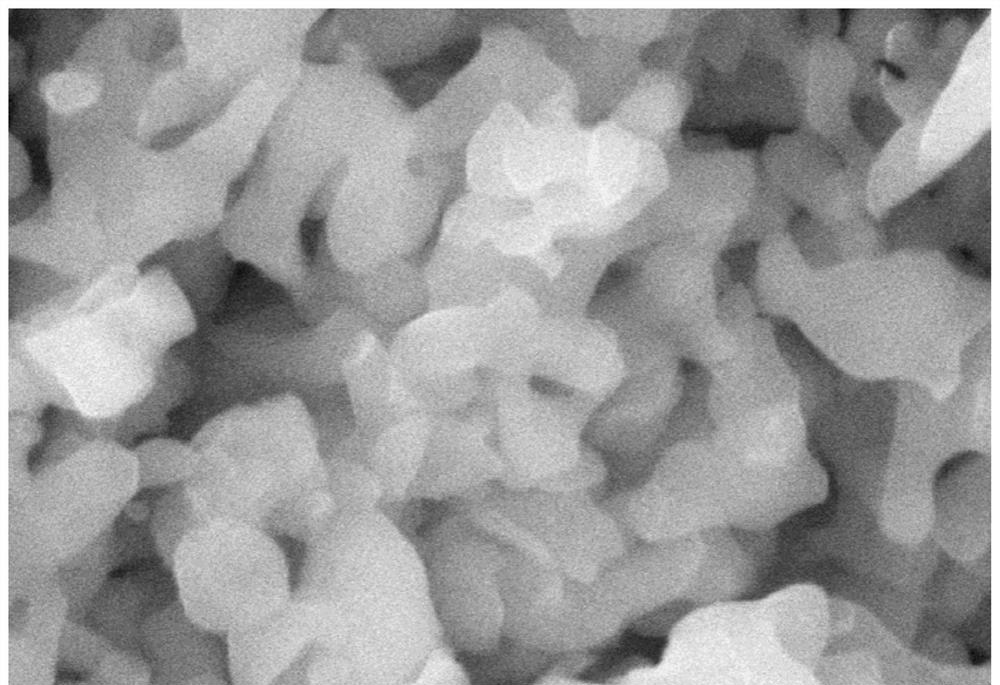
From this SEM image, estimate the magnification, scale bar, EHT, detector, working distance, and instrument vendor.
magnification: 100 K X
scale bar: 500 nm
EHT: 10 kV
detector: SE(UL)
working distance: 10.5 mm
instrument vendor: Hitachi